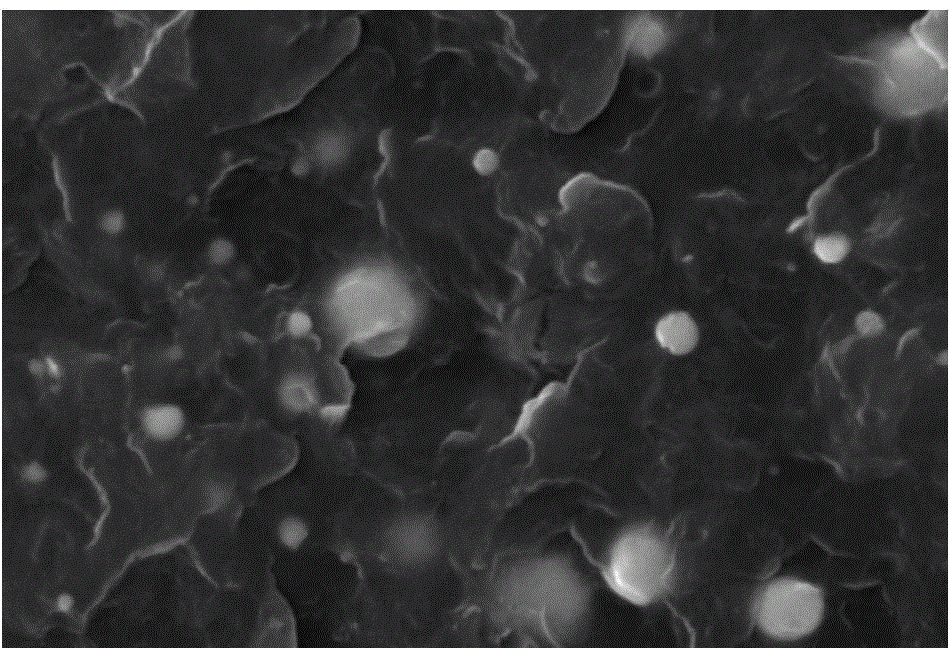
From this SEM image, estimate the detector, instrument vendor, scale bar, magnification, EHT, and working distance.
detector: SEI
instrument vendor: JEOL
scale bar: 10000 nm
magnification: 2 K X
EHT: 30 kV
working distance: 11 mm